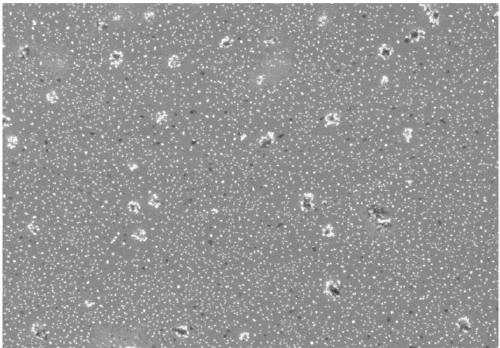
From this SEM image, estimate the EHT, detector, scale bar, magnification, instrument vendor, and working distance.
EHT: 3 kV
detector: SE(UL)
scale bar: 10000 nm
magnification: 5 K X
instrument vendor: Hitachi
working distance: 8.2 mm